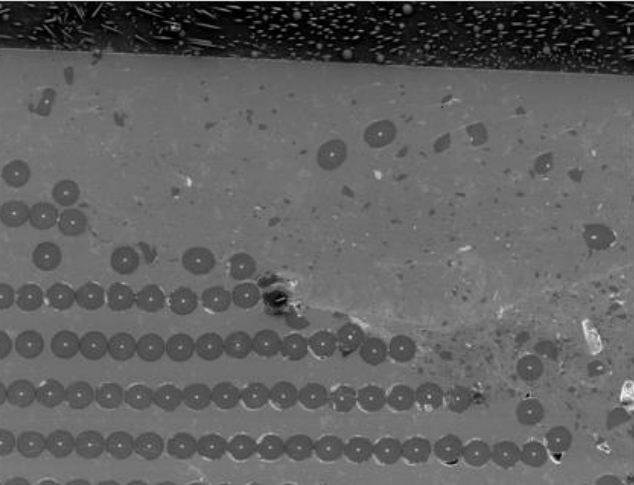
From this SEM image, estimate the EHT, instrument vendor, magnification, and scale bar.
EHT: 15 kV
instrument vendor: JEOL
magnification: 0.031 K X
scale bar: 322000 nm